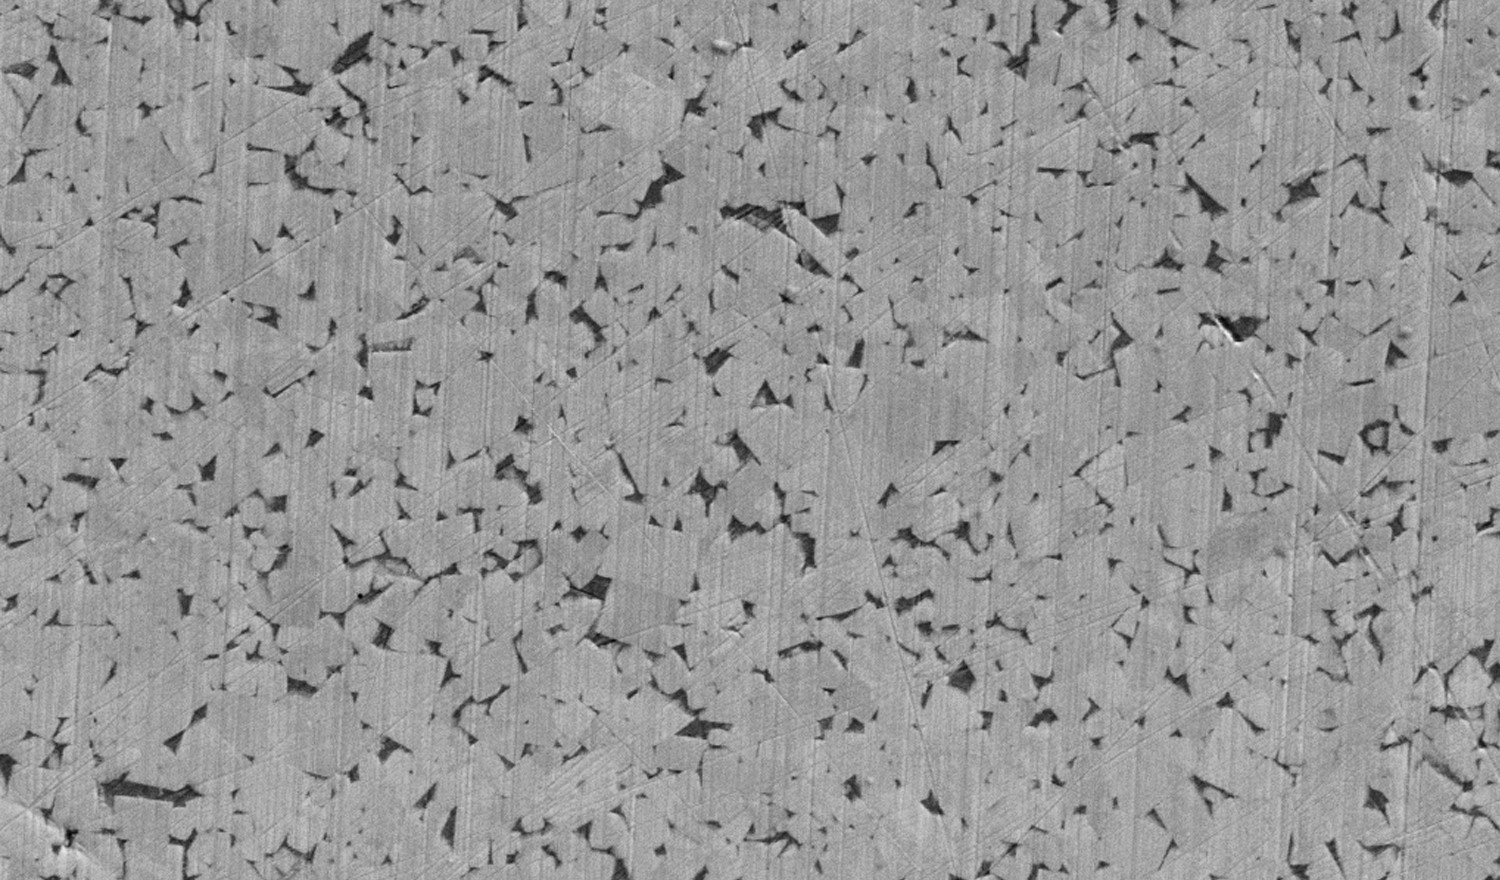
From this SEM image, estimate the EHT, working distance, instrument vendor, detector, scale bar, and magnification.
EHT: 5 kV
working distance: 6.9 mm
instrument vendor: Zeiss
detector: SE2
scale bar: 2000 nm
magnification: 5 K X